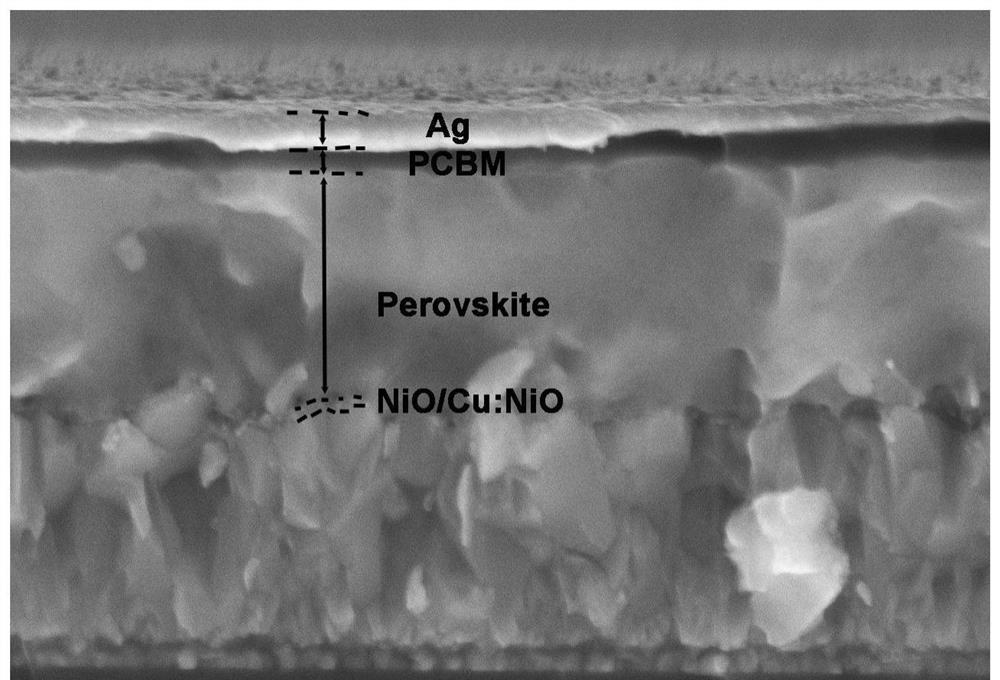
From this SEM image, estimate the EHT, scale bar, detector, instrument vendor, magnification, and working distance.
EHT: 10 kV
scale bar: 1000 nm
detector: SE(U)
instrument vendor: Hitachi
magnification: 50 K X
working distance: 9 mm